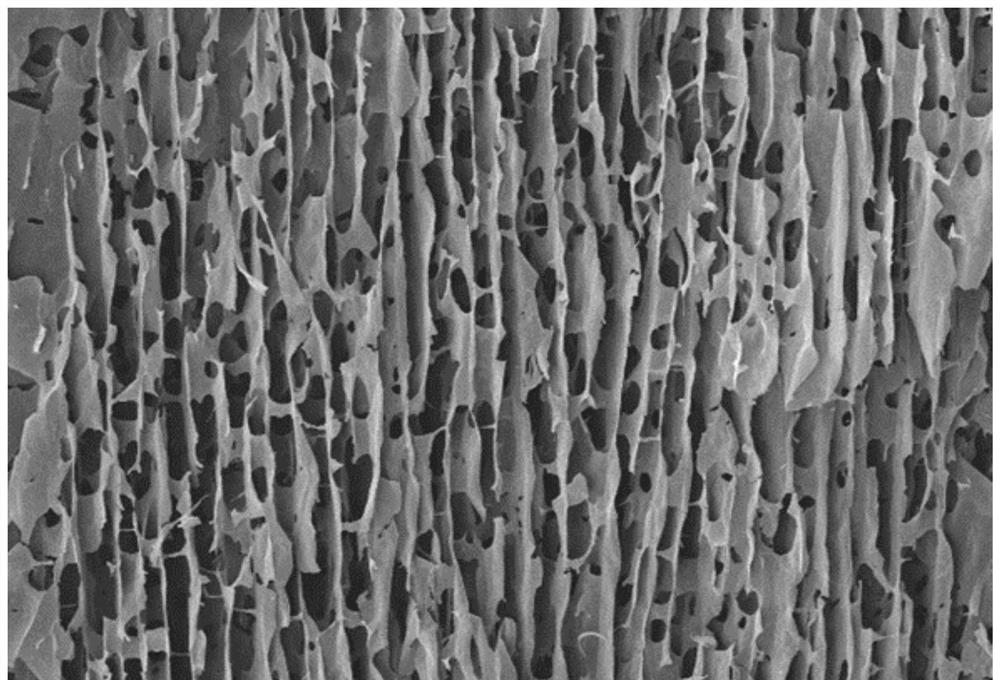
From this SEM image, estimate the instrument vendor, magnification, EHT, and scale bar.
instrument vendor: JEOL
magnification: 0.3 K X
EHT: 20 kV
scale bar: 50000 nm